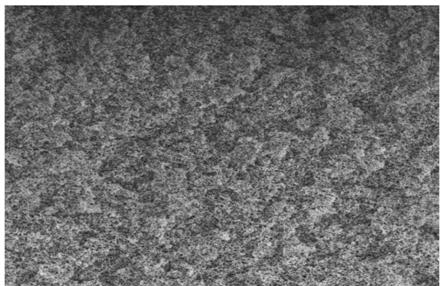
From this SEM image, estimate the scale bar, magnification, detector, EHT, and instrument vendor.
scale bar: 30000 nm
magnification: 1.5 K X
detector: SE(M)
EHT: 10 kV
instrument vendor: Hitachi